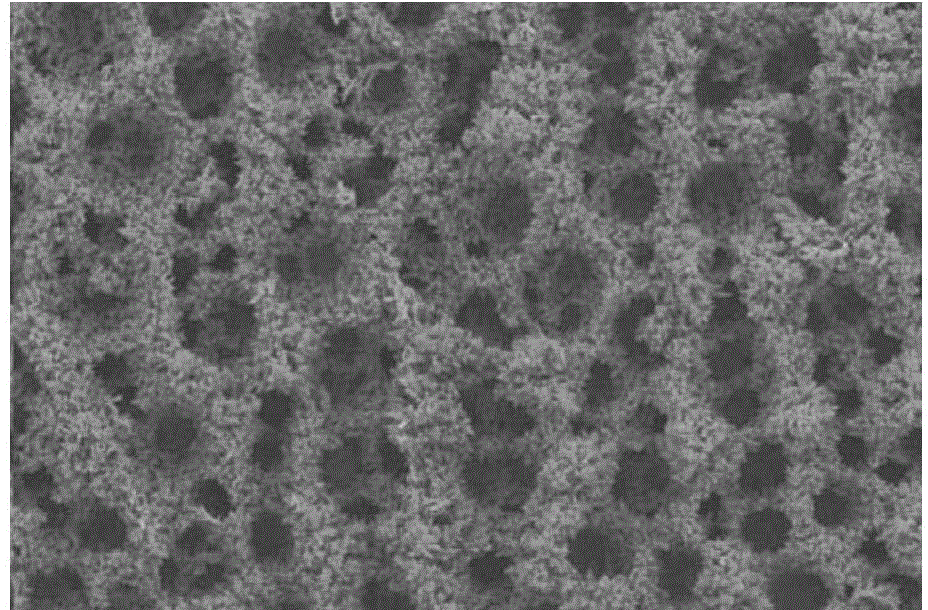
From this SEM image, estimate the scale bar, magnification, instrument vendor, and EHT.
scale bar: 500000 nm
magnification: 0.1 K X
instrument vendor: JEOL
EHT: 15 kV